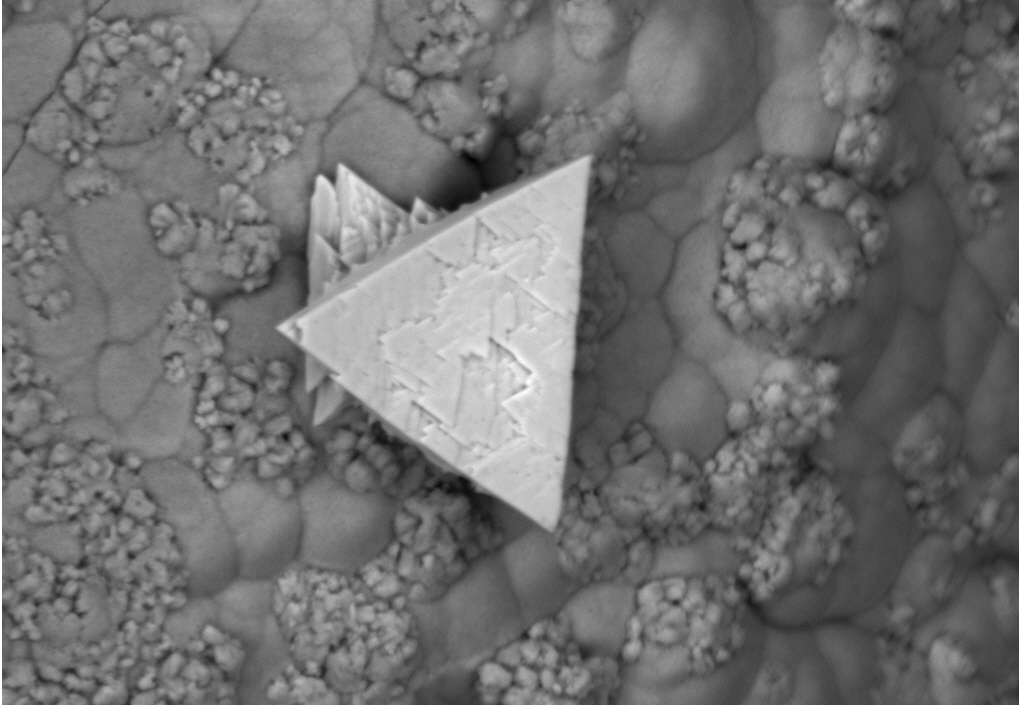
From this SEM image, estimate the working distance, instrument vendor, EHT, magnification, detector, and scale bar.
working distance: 9.5 mm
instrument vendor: Zeiss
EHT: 15 kV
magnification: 1.38 K X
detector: NTS BSD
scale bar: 10000 nm